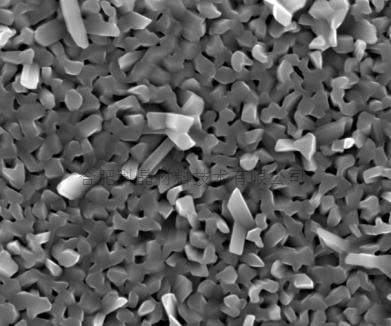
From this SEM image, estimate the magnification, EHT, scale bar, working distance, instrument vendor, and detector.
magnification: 20 K X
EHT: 20 kV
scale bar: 2000 nm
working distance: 6 mm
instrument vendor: FEI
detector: TLD